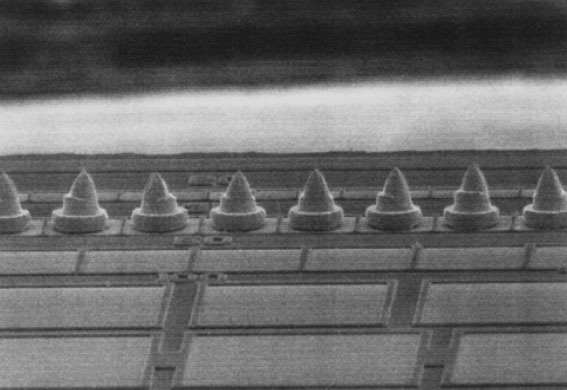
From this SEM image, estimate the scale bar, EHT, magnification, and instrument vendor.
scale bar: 50000 nm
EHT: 15 kV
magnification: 0.5 K X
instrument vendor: JEOL